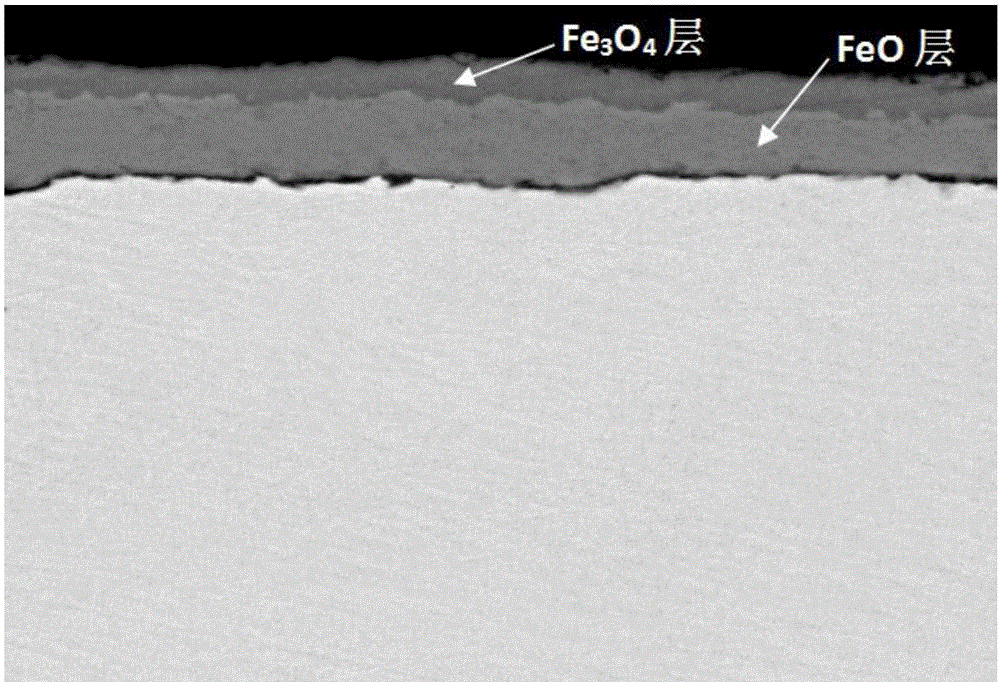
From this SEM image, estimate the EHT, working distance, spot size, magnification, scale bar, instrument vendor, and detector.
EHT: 20 kV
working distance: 11.9 mm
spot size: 4.5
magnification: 5 K X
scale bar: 10000 nm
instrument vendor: FEI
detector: SSD